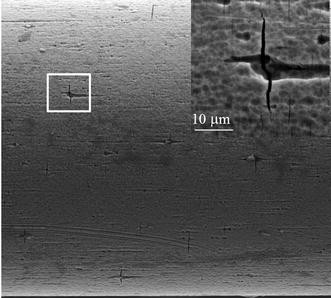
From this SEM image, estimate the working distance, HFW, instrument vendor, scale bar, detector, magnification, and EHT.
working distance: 24.82 mm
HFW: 212 µm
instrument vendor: Tescan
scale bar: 50000 nm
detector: SE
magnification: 0.98 K X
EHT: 20 kV